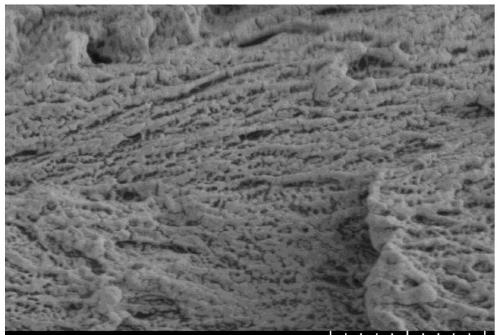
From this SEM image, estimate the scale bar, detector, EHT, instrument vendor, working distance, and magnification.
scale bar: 2000 nm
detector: SE(L)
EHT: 2 kV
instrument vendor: Hitachi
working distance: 12.3 mm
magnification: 20 K X